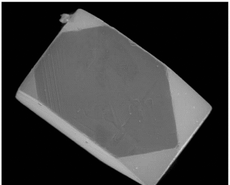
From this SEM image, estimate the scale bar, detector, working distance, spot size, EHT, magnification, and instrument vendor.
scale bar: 50000 nm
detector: LFD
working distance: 9.7 mm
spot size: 5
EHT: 30 kV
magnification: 0.8 K X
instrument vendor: FEI